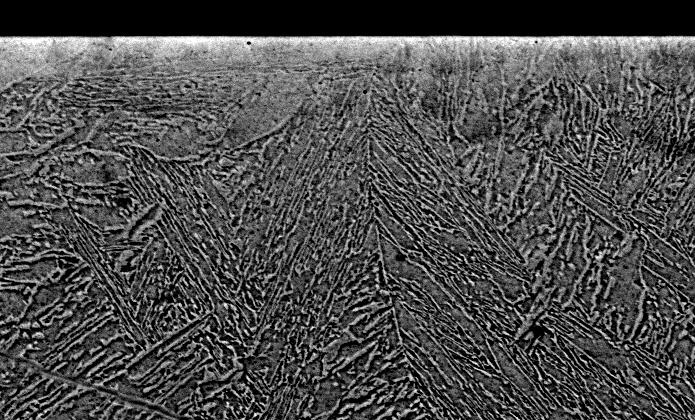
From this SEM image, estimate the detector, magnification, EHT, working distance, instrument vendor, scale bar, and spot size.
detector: BSE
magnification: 1.5 K X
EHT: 20 kV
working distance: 10.9 mm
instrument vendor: FEI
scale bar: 20000 nm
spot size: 5